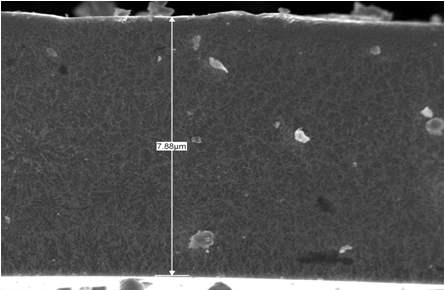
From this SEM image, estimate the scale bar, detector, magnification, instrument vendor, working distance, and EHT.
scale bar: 1000 nm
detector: SEI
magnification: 10 K X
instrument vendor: JEOL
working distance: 8.1 mm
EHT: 5 kV